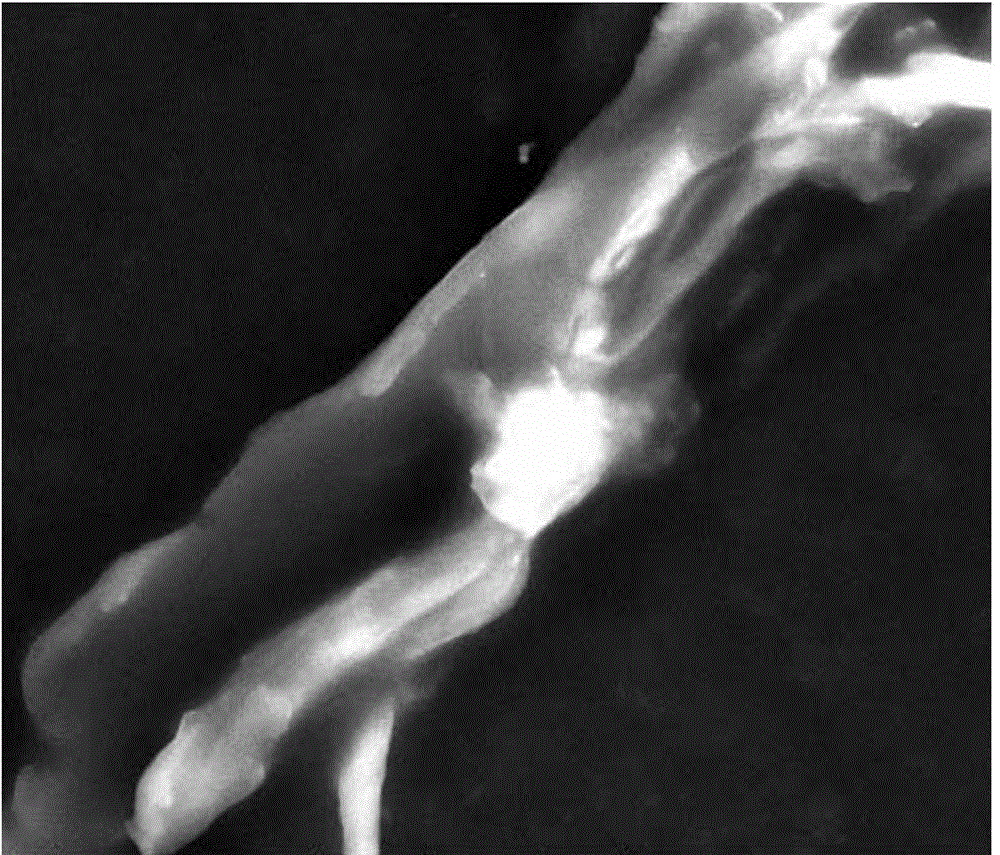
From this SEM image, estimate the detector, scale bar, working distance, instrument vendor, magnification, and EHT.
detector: SE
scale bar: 10000 nm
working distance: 5.9 mm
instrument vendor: FEI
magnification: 6 K X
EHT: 20 kV